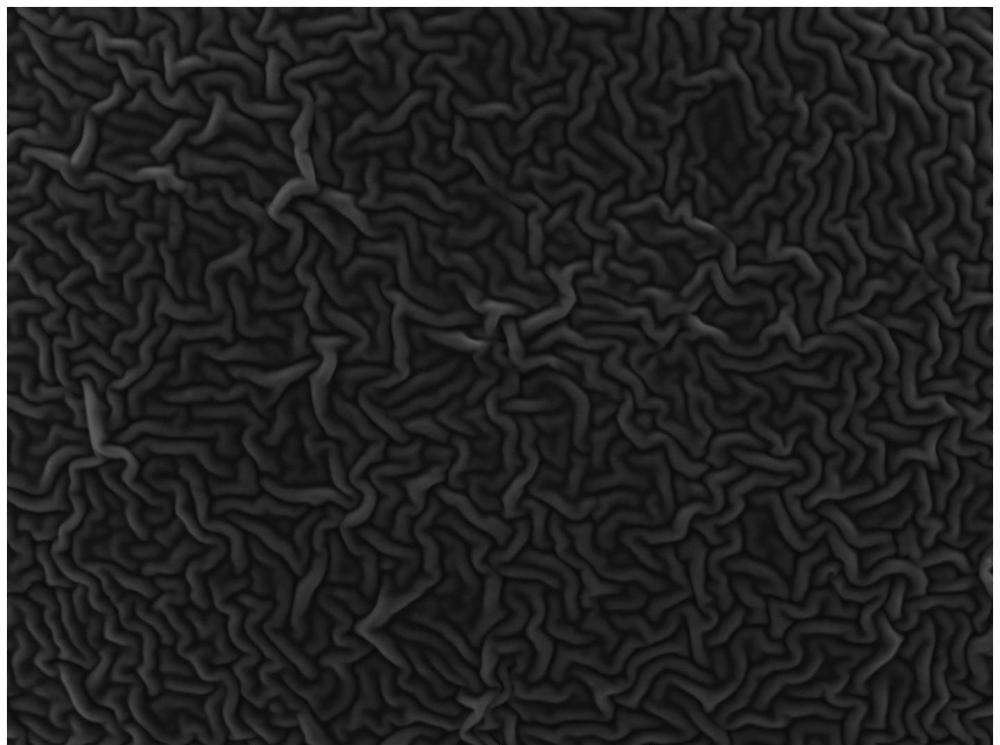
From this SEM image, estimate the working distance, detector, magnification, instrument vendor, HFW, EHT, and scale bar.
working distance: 22.93 mm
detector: SE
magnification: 2.14 K X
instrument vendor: Tescan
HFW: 130 µm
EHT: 10 kV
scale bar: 20000 nm